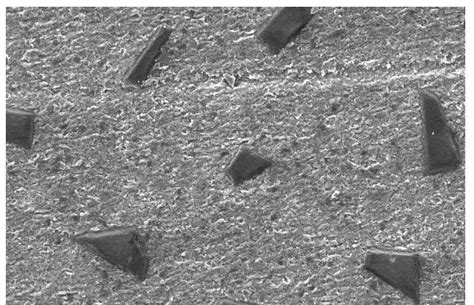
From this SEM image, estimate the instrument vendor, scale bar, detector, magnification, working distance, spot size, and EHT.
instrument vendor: FEI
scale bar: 100000 nm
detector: SE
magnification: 0.5 K X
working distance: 9.9 mm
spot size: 4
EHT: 20 kV